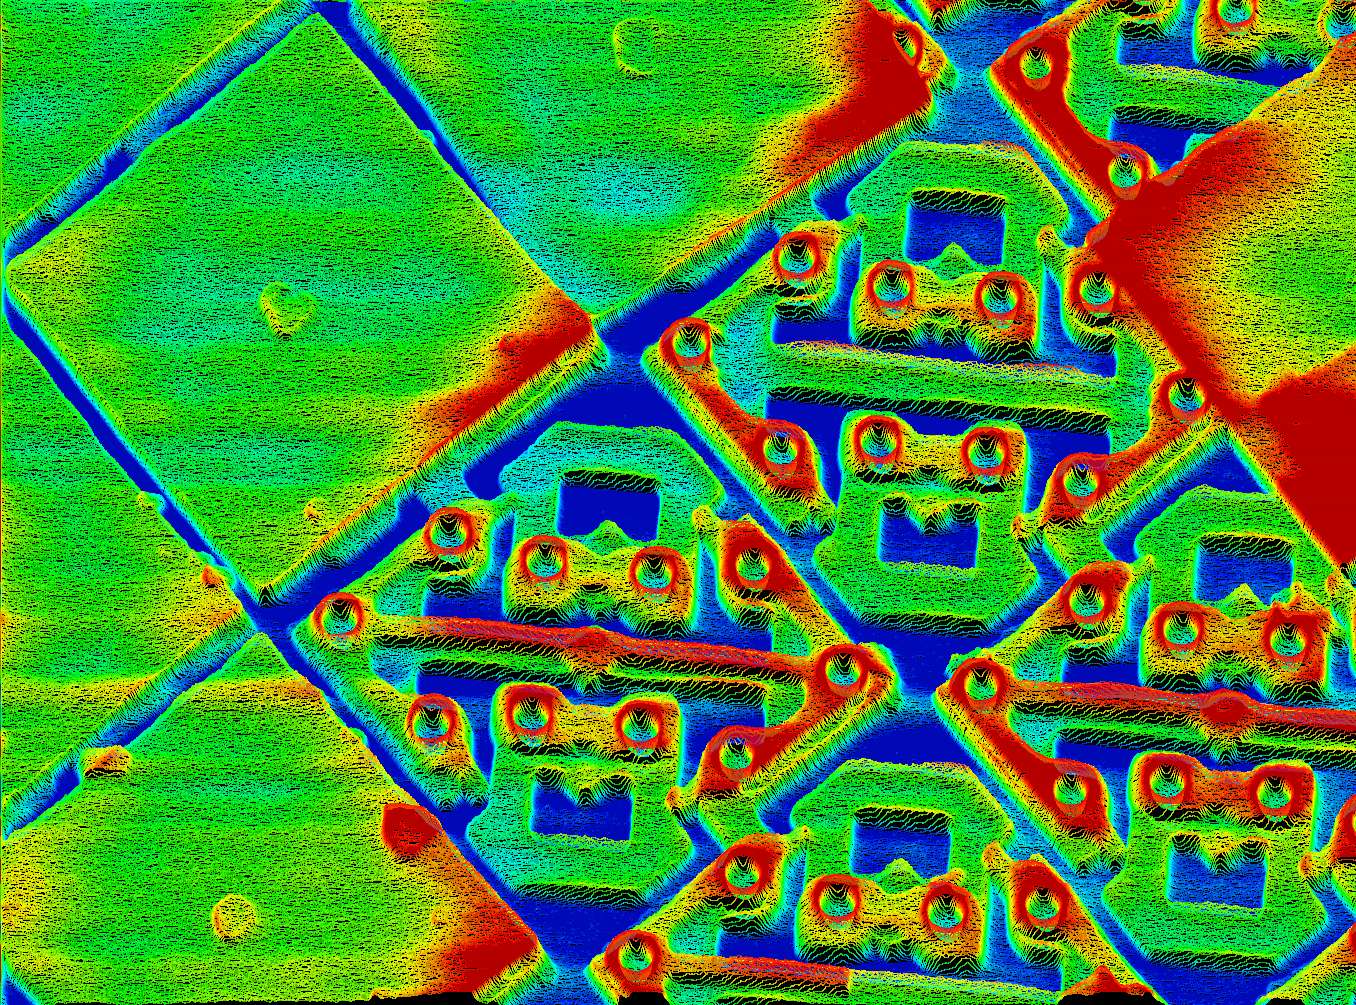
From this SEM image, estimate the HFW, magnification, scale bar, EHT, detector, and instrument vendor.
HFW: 40 µm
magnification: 3 K X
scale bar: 3330 nm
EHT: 15 kV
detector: SE +Y-МОД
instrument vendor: other